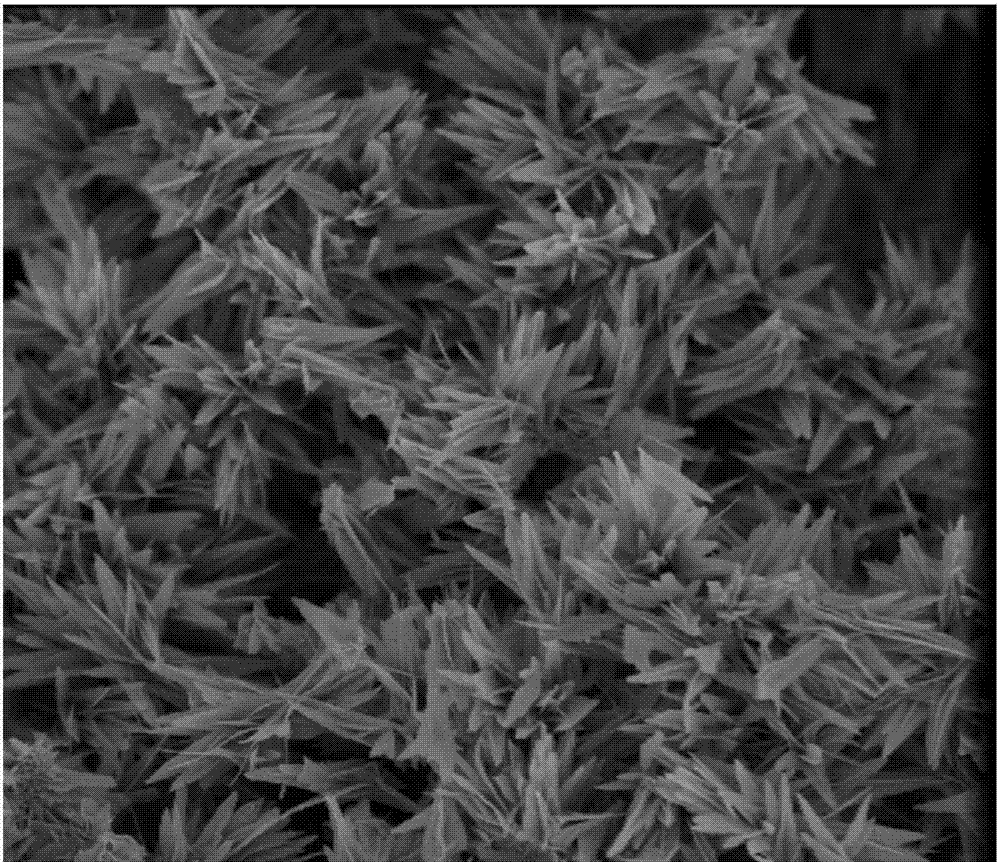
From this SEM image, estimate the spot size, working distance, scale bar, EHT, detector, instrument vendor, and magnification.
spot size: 3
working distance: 5.6 mm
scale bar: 5000 nm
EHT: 3 kV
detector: TLD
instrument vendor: FEI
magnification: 10 K X